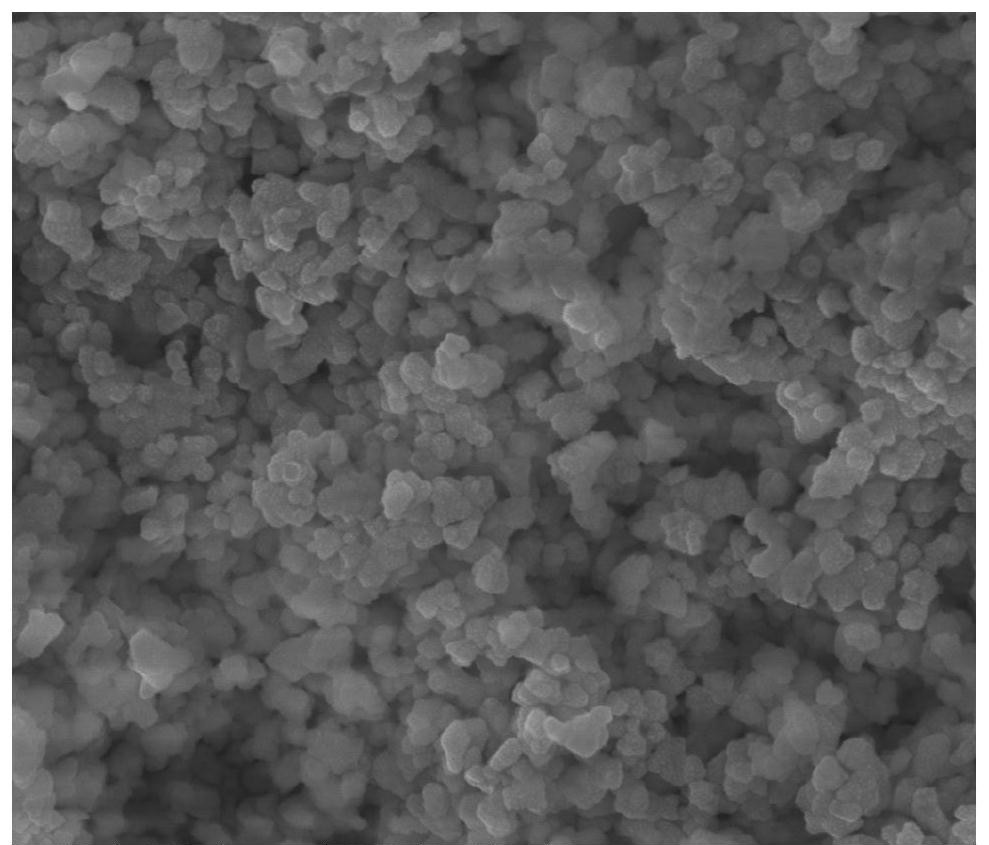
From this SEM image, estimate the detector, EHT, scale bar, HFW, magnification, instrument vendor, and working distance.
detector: ETD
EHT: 20 kV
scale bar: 2000 nm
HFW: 5.97 µm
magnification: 50 K X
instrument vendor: FEI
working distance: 10.2 mm